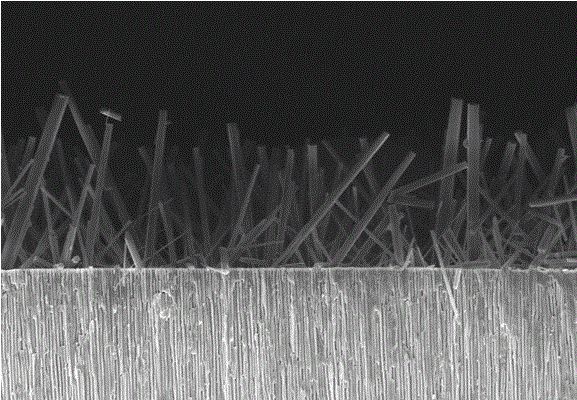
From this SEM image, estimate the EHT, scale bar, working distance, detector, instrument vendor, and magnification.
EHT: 5 kV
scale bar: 20000 nm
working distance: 9 mm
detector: SE(U)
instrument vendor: Hitachi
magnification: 2 K X